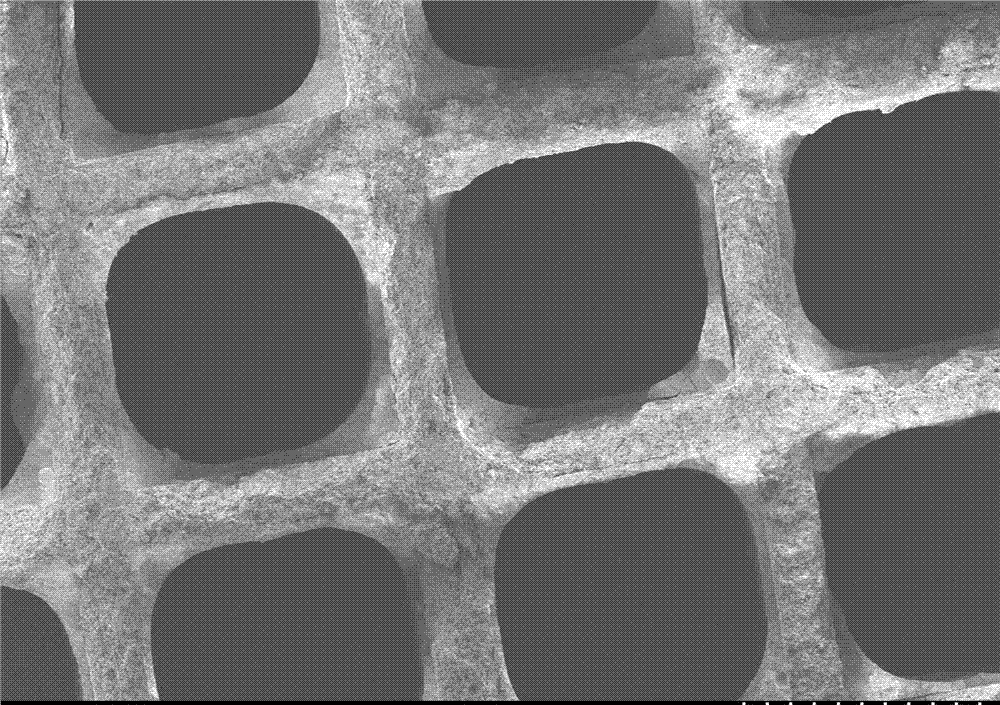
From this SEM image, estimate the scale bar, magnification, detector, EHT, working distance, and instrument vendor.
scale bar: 1000000 nm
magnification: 0.03 K X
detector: SE(M)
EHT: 5 kV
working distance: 13.4 mm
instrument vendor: Hitachi